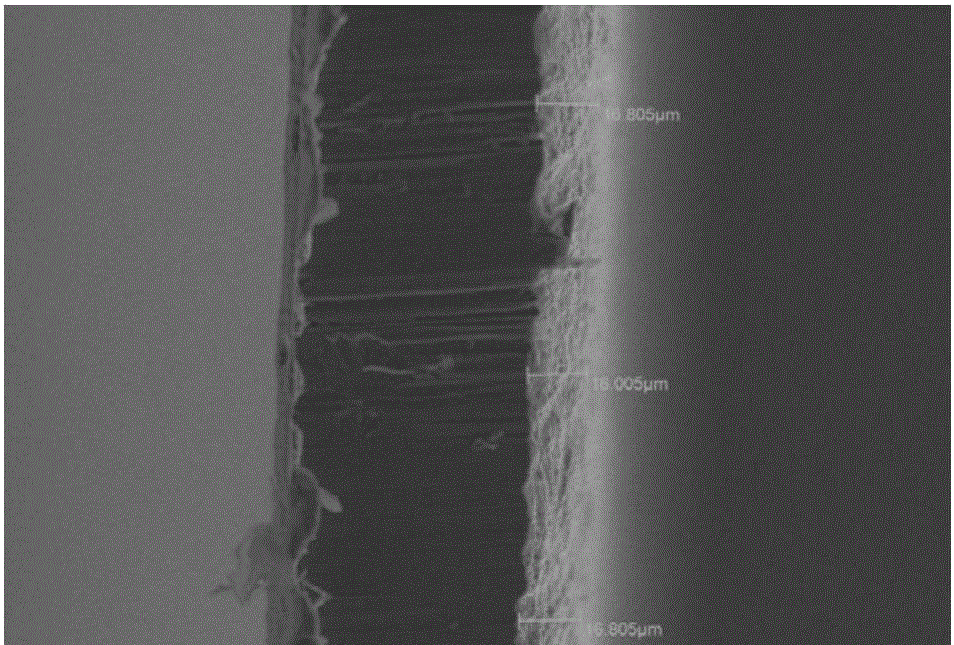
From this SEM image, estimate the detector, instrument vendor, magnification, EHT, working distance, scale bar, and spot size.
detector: SEI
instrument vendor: JEOL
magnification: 0.5 K X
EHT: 25 kV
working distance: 4 mm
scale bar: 50000 nm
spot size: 30